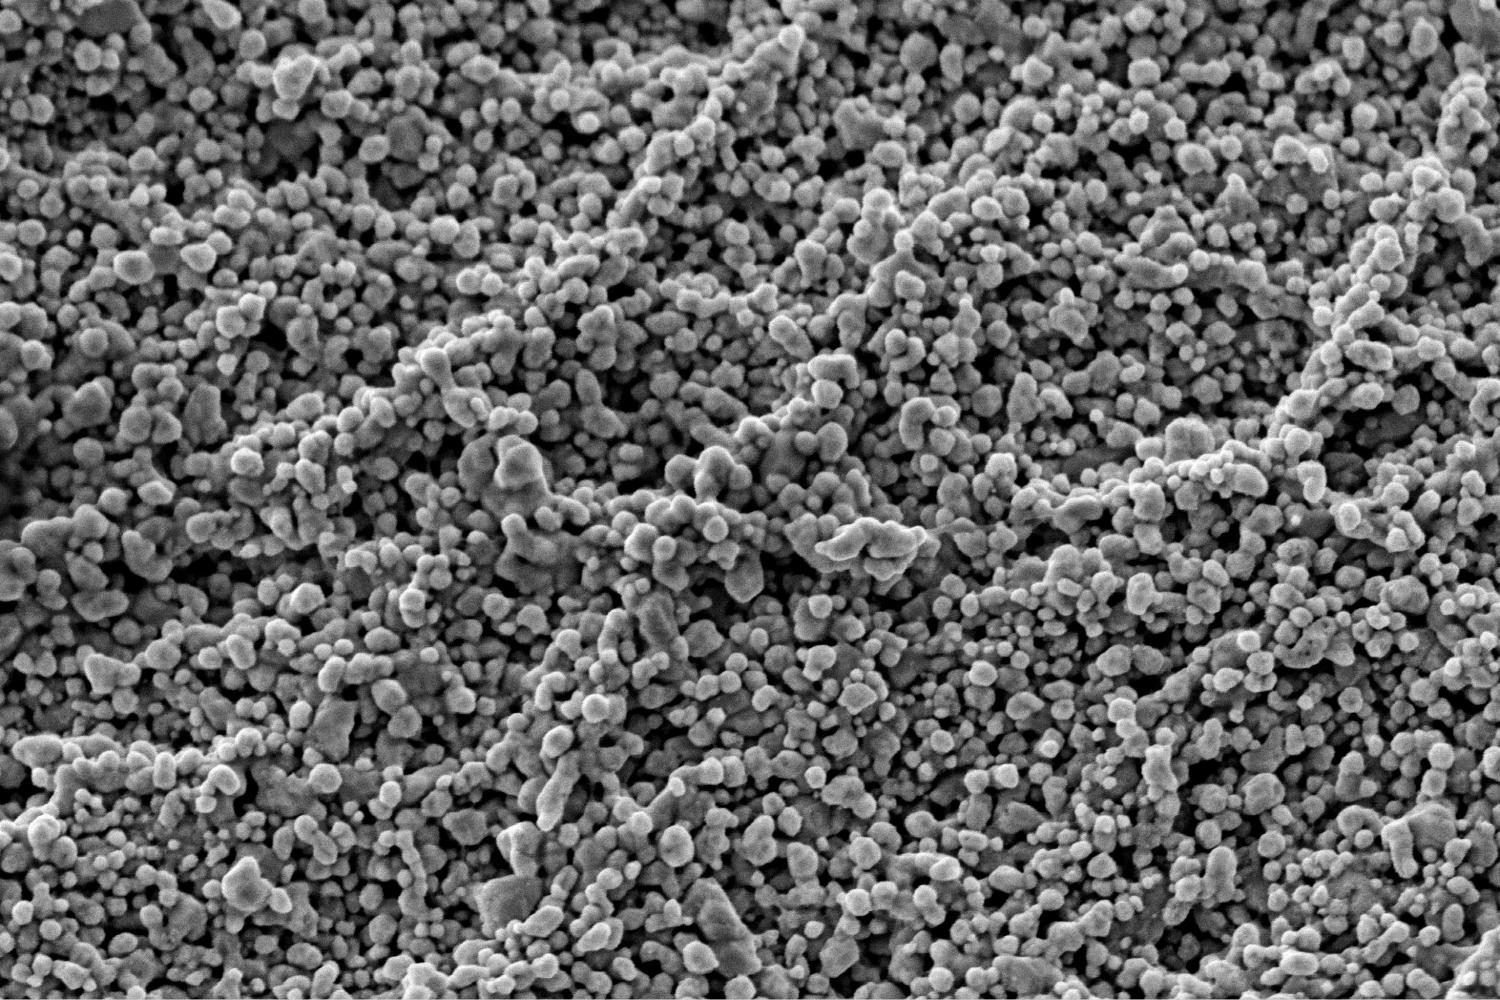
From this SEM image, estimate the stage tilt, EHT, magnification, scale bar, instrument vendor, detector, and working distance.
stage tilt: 0°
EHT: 2 kV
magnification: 150 K X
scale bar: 500 nm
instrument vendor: FEI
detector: TLD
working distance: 3.8 mm